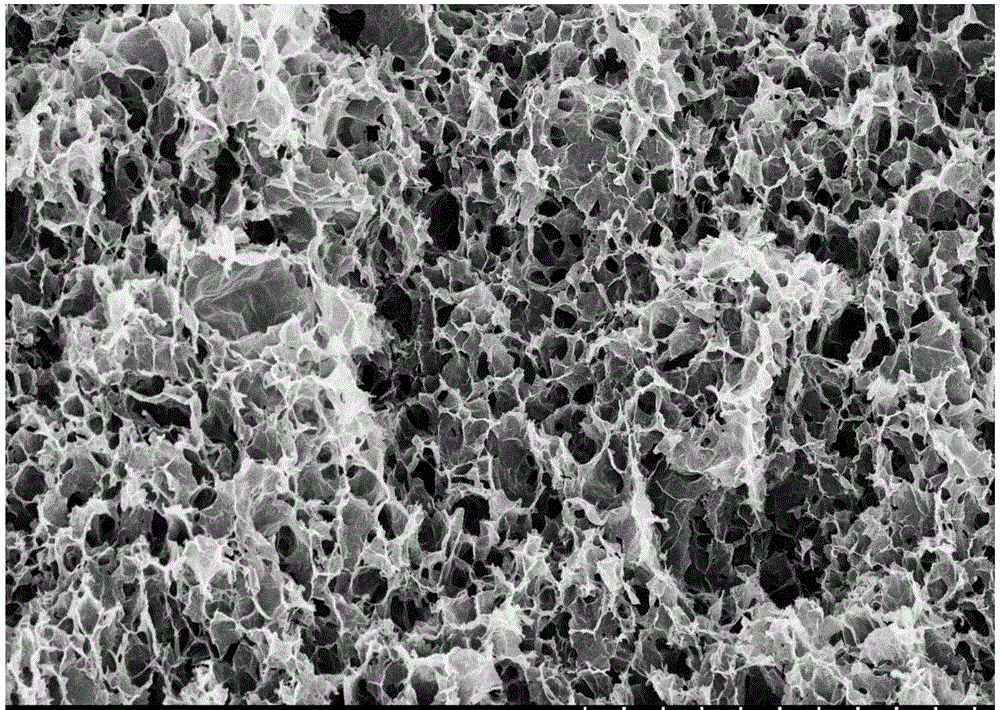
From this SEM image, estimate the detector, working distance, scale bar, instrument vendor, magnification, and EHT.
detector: SE(M,LA0)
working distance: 8.5 mm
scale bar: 50000 nm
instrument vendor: Hitachi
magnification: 1 K X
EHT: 5 kV